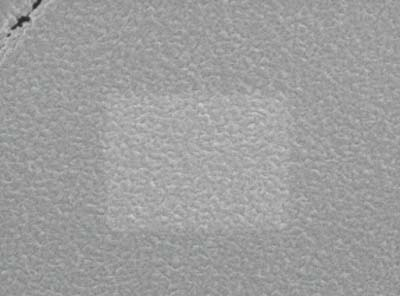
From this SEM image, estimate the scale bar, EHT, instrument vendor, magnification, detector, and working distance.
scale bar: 100 nm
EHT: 1 kV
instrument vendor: JEOL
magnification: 50 K X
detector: SEI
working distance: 3 mm